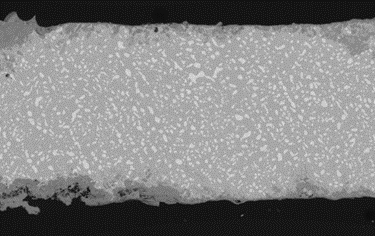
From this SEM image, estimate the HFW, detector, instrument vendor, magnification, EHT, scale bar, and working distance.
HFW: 1260 µm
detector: BSE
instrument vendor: Tescan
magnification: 0.22 K X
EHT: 20 kV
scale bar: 200000 nm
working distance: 15 mm